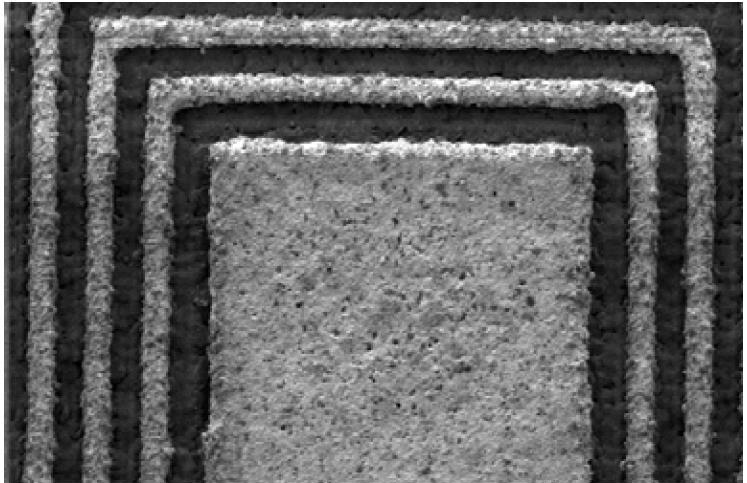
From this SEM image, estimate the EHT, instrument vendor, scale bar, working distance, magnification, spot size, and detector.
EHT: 1.1 kV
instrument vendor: FEI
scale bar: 50000 nm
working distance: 10.3 mm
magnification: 0.5 K X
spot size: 3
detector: SE